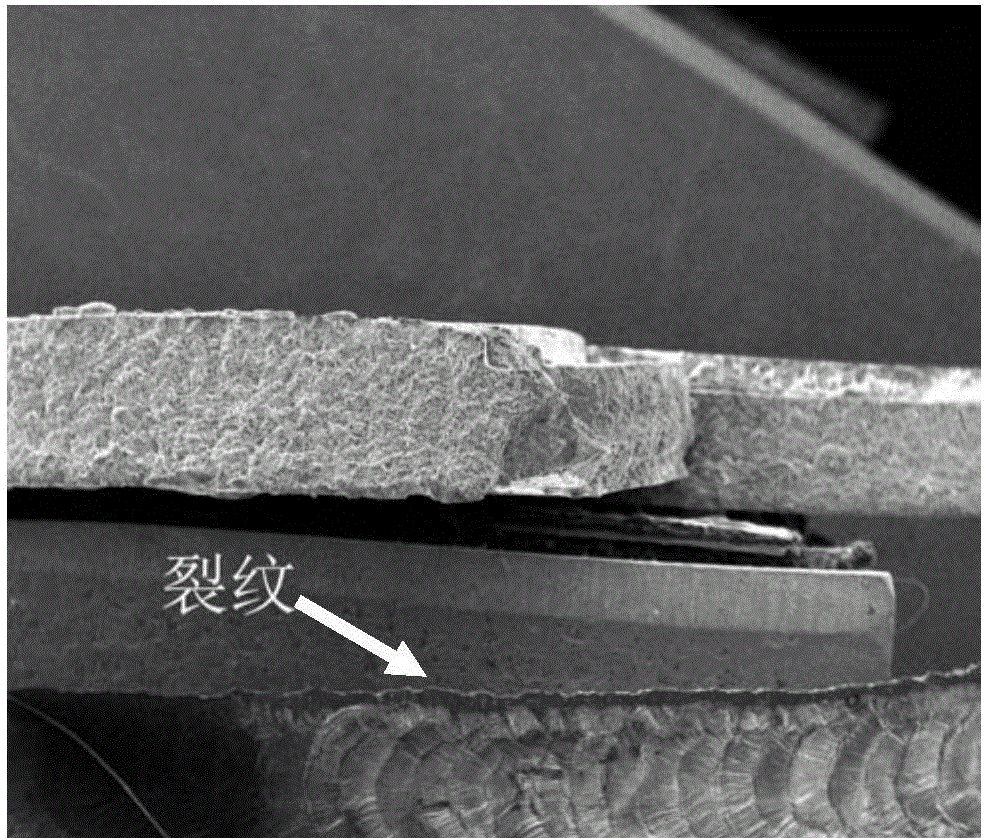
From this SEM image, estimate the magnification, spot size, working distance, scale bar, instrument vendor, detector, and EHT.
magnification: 0.05 K X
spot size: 5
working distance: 18.2 mm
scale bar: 3000000 nm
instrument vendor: FEI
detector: ETD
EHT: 20 kV